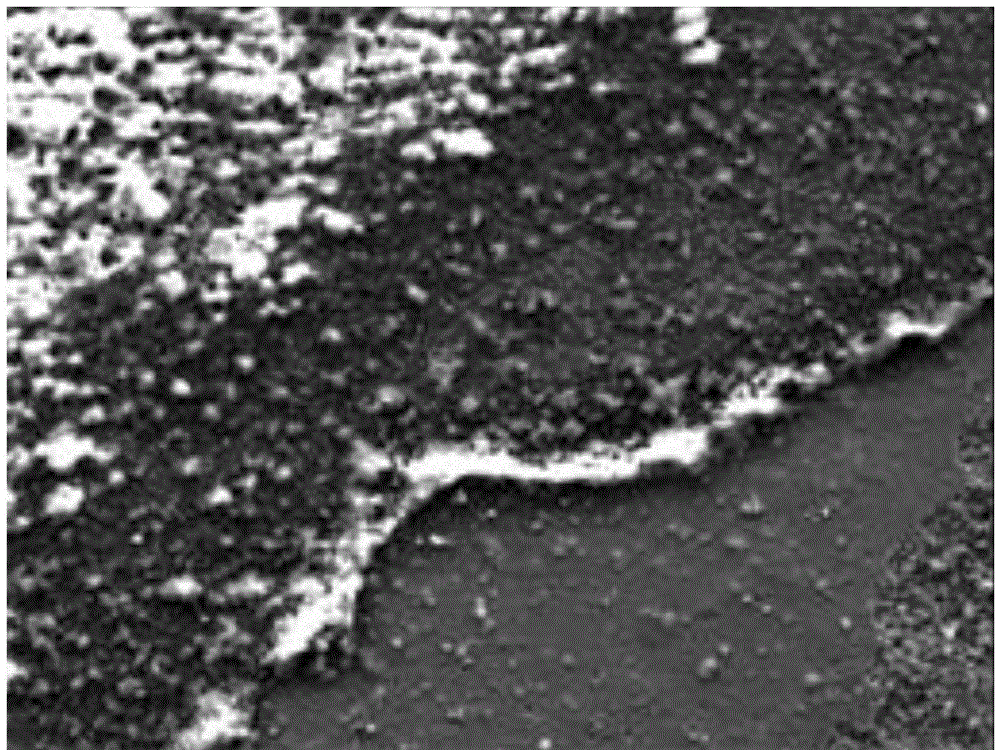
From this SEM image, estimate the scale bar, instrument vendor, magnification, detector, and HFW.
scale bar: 200000 nm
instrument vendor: Tescan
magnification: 0.3 K X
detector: SE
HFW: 692 µm